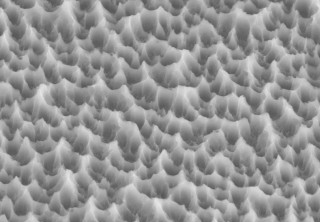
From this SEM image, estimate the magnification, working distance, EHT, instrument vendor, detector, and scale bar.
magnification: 13 K X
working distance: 19.4 mm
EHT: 7 kV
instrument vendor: Hitachi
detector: SE(M)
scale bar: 4000 nm